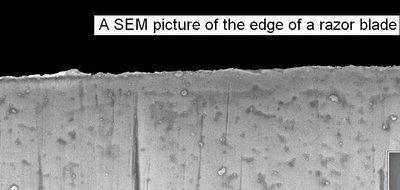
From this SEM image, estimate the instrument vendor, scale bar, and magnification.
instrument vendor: Zeiss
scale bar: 1000 nm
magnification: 25 K X